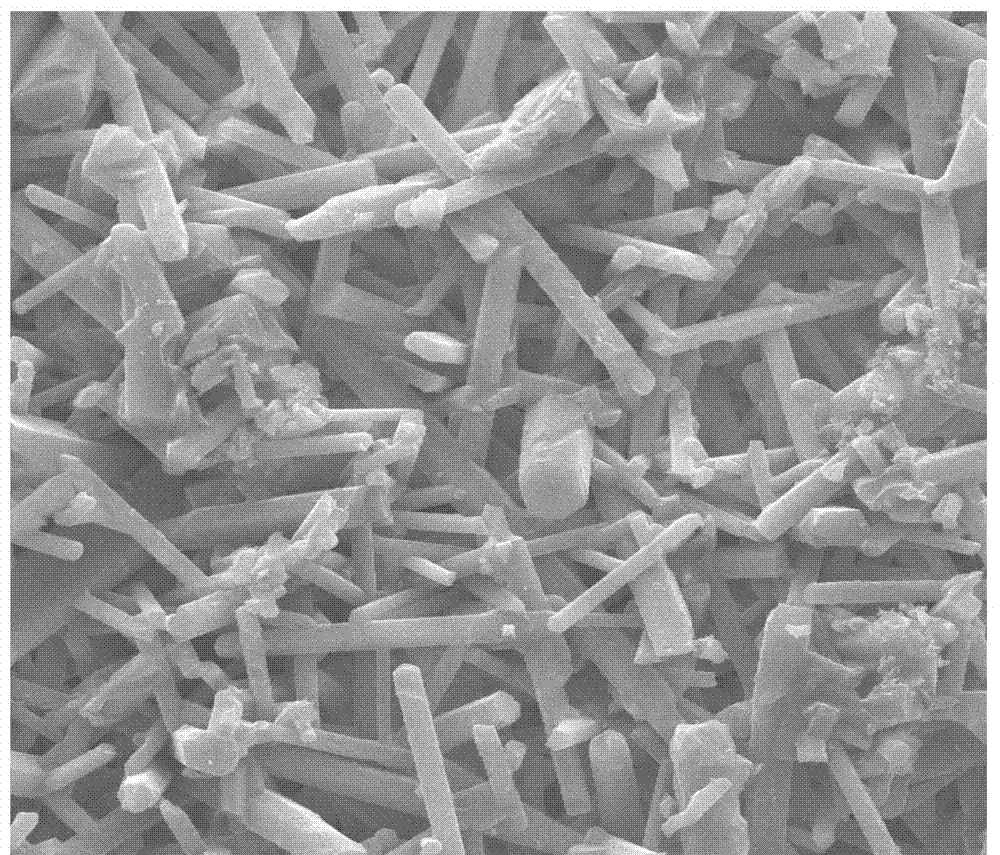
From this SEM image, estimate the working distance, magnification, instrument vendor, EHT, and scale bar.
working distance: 10.6 mm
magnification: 5 K X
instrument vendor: FEI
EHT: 20 kV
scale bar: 20000 nm